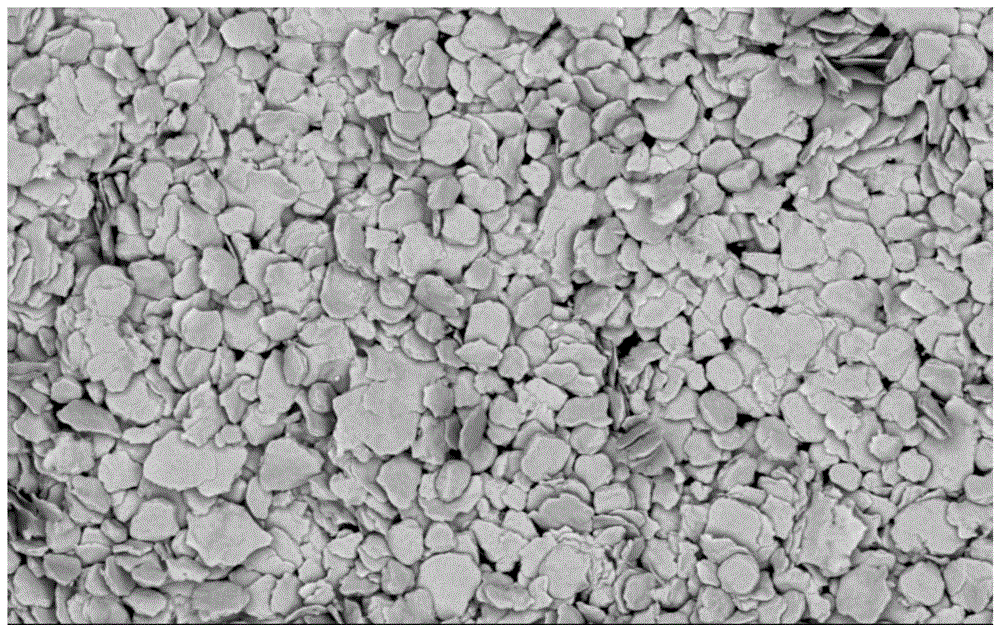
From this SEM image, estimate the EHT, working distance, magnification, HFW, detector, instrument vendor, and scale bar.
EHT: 10 kV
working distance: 7.641 mm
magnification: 8 K X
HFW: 64.9 µm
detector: BSD Full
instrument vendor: Thermo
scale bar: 20000 nm